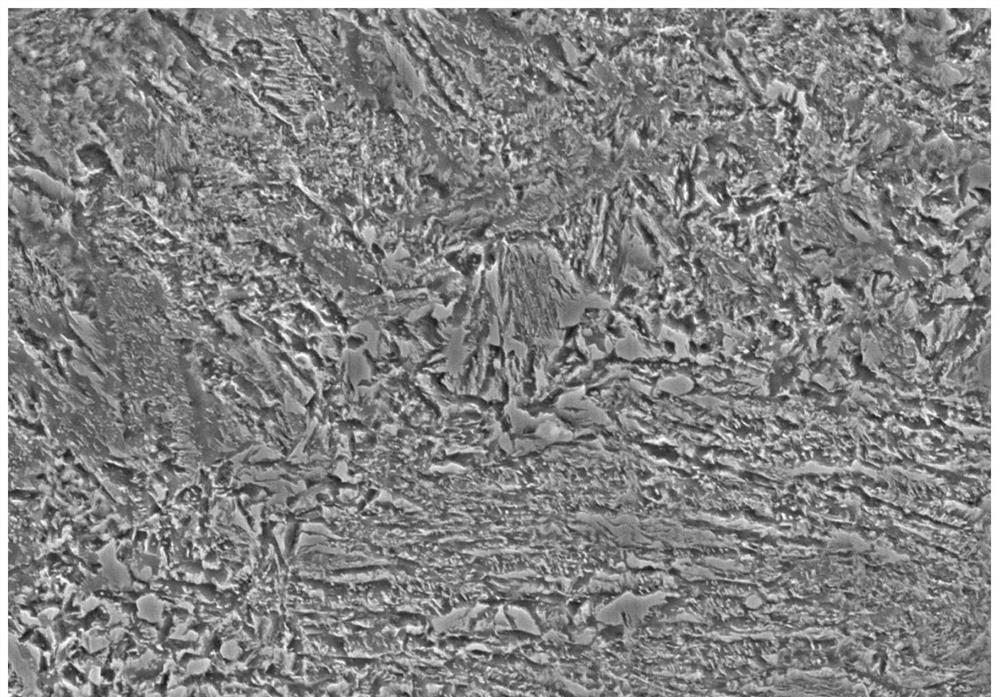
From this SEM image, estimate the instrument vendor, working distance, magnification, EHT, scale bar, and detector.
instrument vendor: Hitachi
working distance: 8.4 mm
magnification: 5 K X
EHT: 15 kV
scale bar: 10000 nm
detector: SE(L)